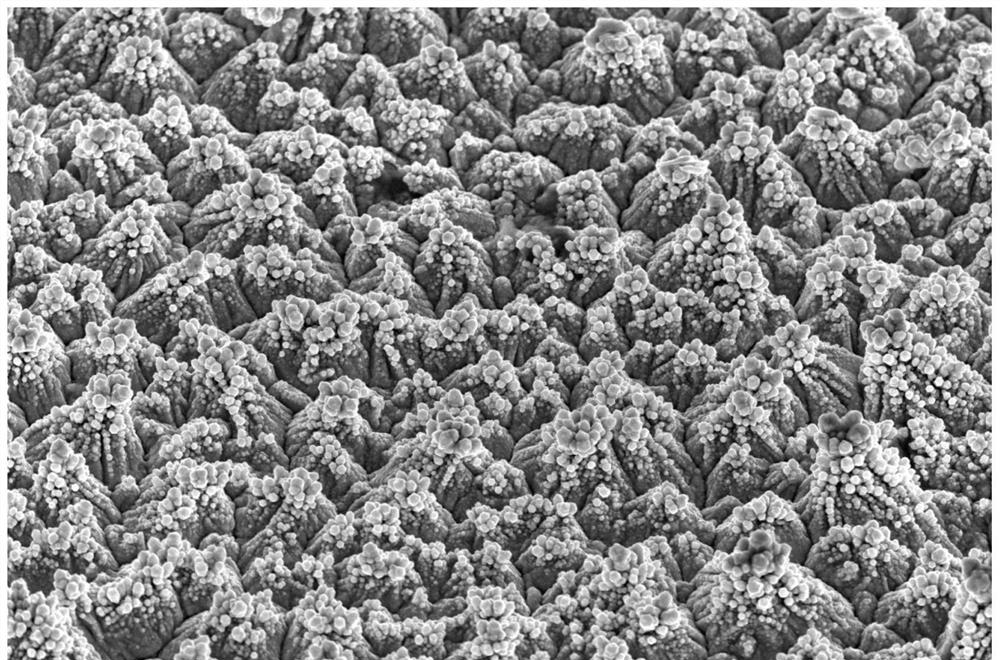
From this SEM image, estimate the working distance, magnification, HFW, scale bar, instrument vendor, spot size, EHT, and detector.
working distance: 13.1 mm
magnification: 5 K X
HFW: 82.9 µm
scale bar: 30000 nm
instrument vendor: FEI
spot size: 3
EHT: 20 kV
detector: ETD